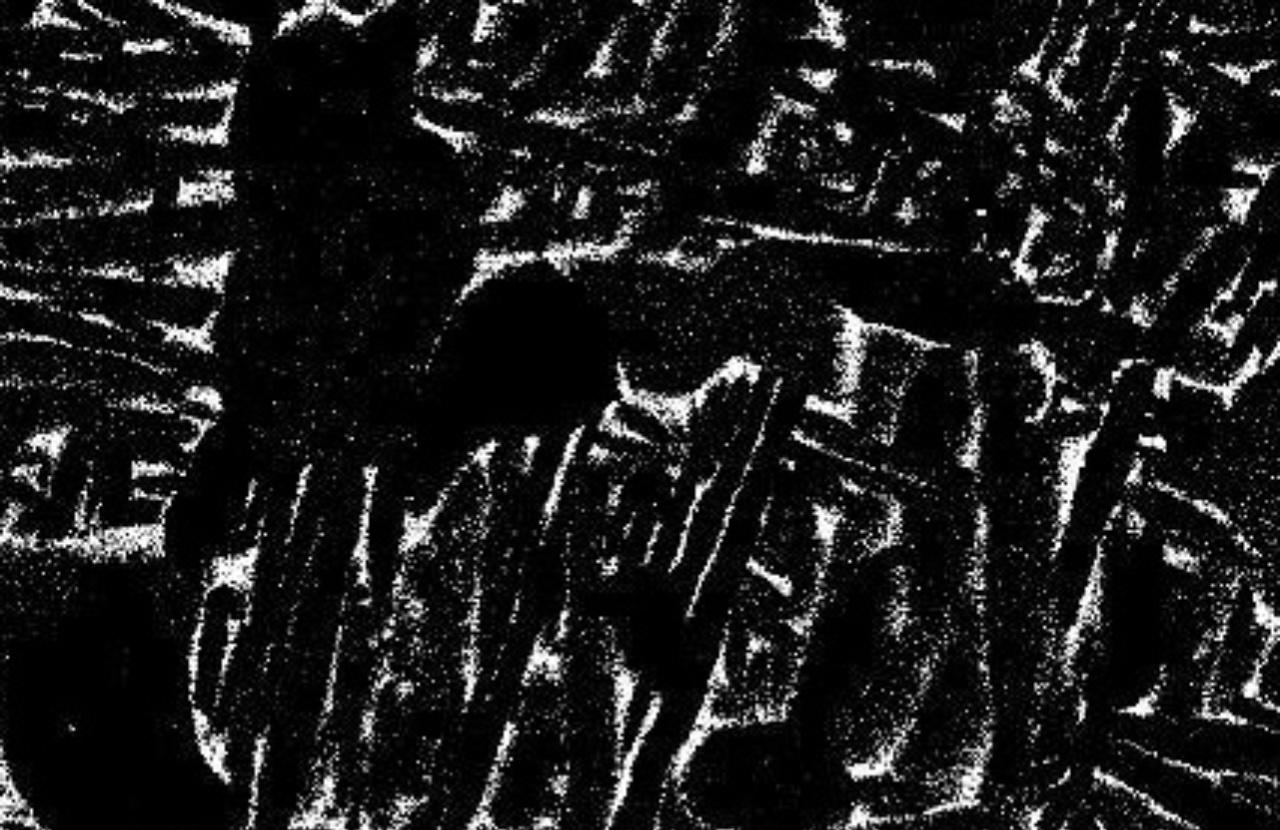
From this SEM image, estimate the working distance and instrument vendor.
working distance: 10.1 mm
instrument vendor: JEOL